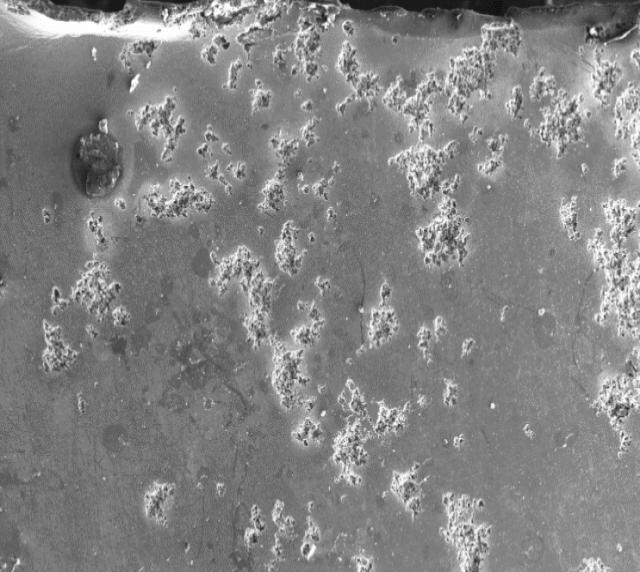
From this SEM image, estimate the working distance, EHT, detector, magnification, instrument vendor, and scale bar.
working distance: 13 mm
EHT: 16 kV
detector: SE1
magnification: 0.1 K X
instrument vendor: Zeiss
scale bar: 200000 nm